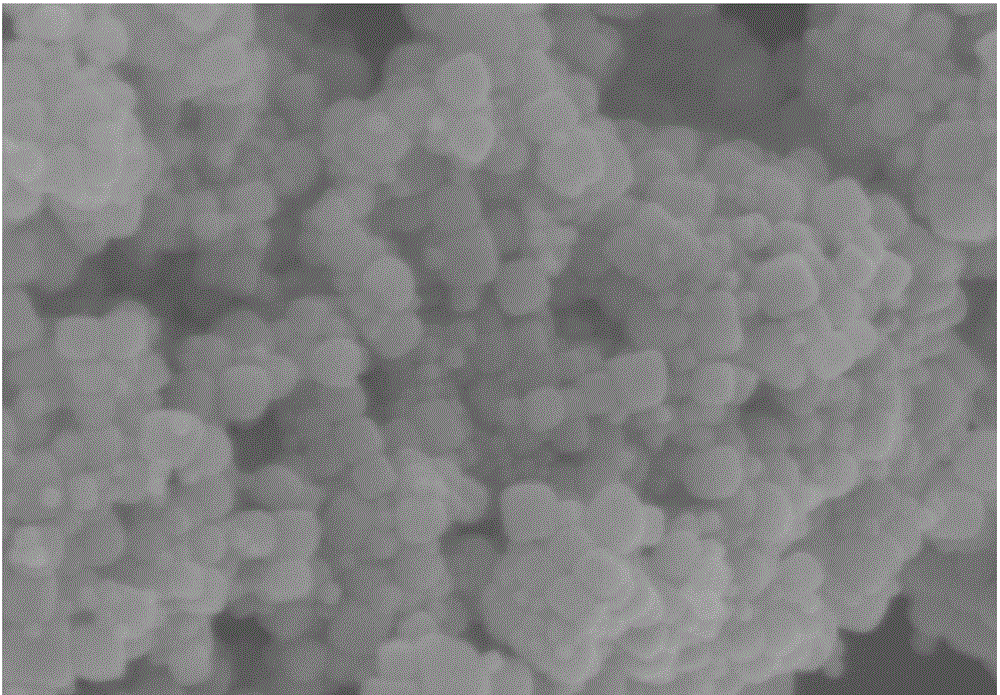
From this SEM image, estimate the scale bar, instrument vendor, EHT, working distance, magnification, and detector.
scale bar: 100 nm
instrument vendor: Zeiss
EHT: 5 kV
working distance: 10 mm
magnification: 100 K X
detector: InLens